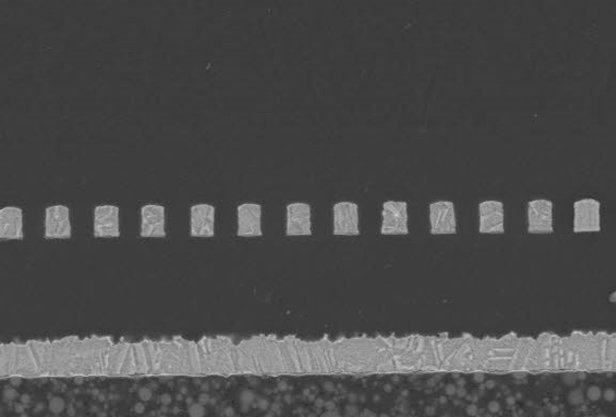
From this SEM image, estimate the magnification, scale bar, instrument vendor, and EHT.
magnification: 3 K X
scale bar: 10000 nm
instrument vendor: JEOL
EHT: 10 kV